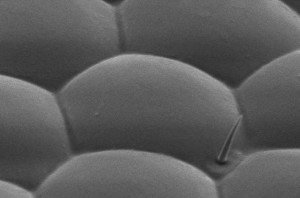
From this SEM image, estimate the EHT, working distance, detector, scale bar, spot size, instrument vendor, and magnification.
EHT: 3 kV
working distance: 4 mm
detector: SEI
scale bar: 5000 nm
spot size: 50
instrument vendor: JEOL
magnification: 5 K X